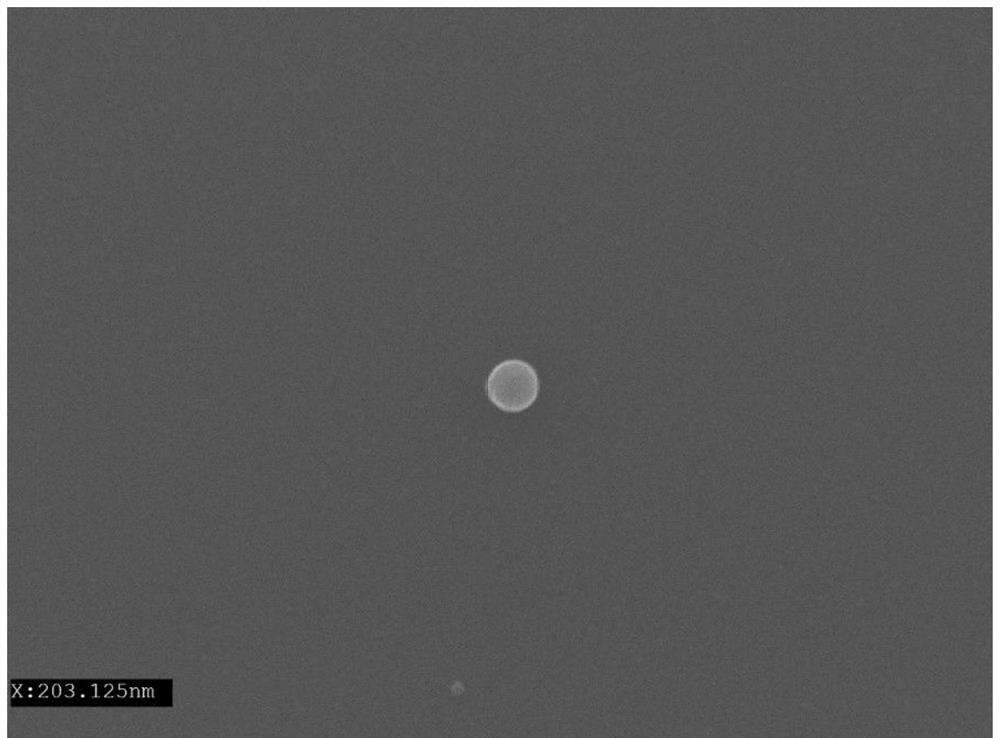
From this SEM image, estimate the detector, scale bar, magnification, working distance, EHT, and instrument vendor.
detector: SEI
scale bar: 100 nm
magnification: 30 K X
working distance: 9.2 mm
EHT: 15 kV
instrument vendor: JEOL